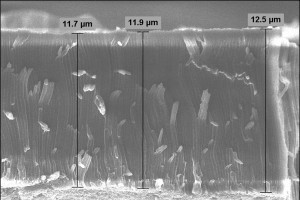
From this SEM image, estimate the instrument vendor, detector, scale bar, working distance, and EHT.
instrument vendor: Zeiss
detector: InLens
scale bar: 3000 nm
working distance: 9.5 mm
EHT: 5 kV